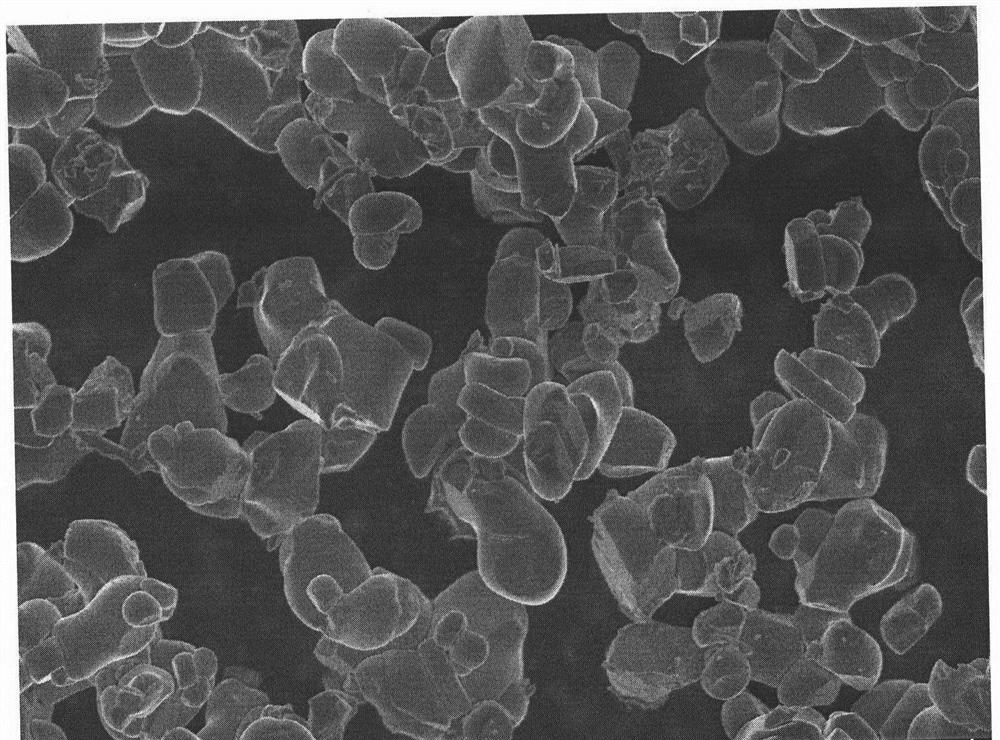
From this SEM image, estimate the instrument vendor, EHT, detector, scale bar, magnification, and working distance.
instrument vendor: Hitachi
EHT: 5 kV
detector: SE(U)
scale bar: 20000 nm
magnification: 2 K X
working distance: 10.7 mm